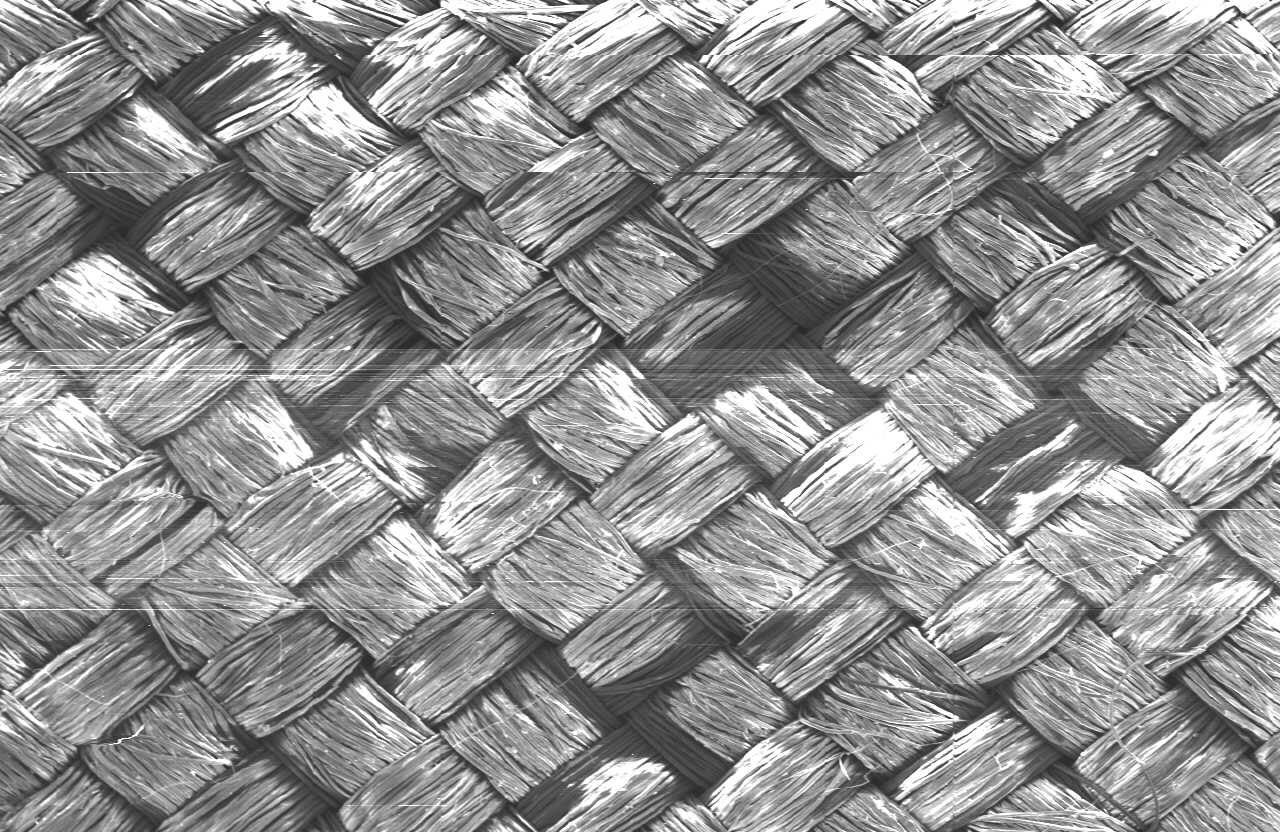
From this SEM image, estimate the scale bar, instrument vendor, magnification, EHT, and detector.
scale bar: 500000 nm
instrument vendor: JEOL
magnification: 0.035 K X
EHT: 13 kV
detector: SEI